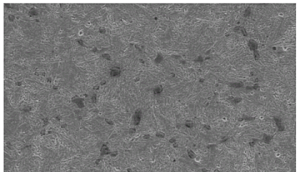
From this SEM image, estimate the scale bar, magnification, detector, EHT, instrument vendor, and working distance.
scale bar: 10000 nm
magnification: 3 K X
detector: SE1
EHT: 15 kV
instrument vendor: Zeiss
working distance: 10.5 mm